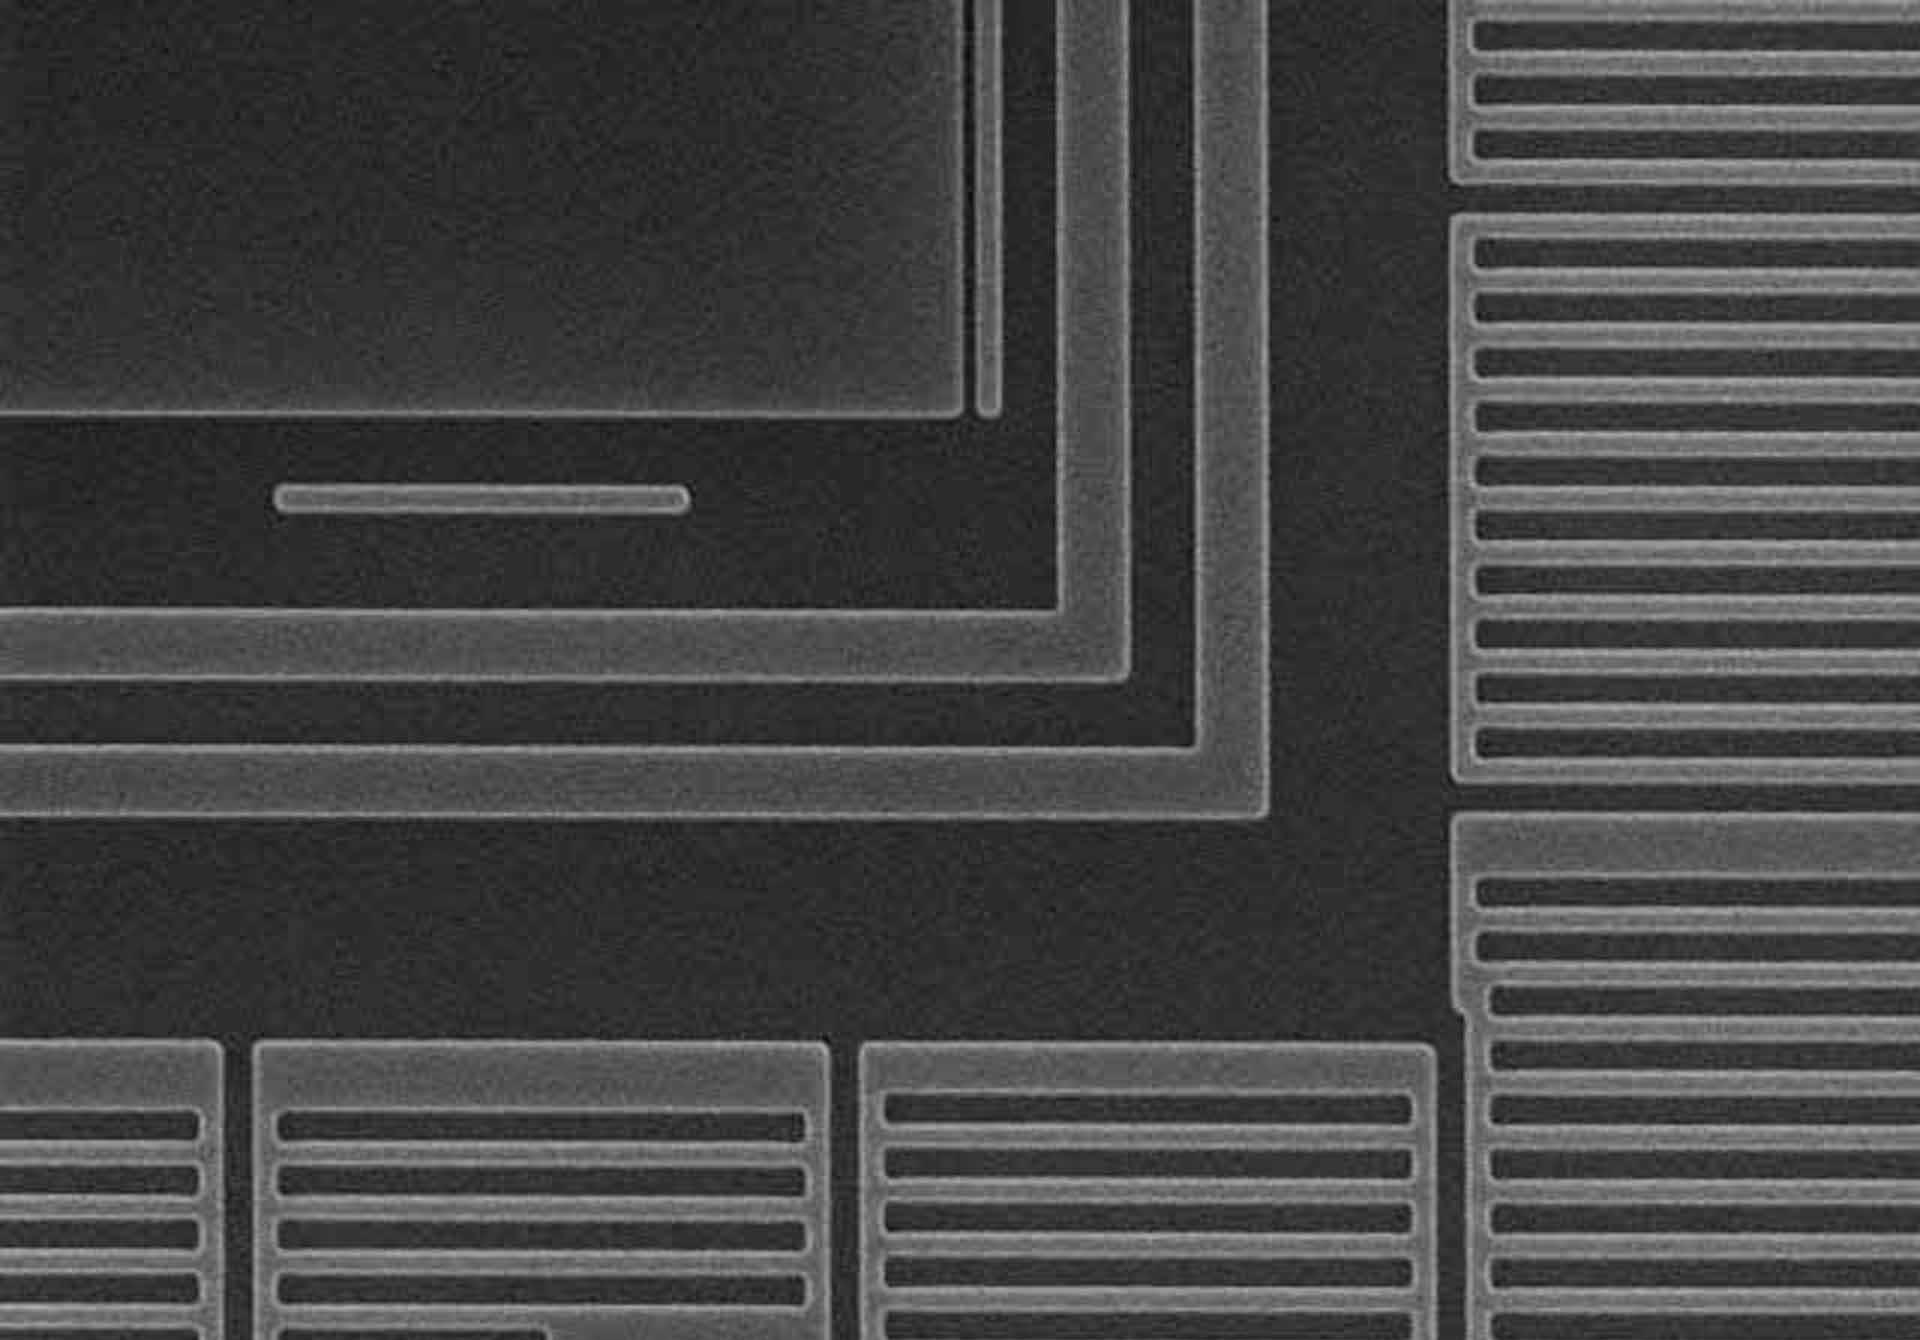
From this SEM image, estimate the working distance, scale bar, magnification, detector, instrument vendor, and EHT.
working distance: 18.8 mm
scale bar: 5000 nm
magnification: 9.04 K X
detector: SE(M)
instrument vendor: Hitachi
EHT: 15 kV